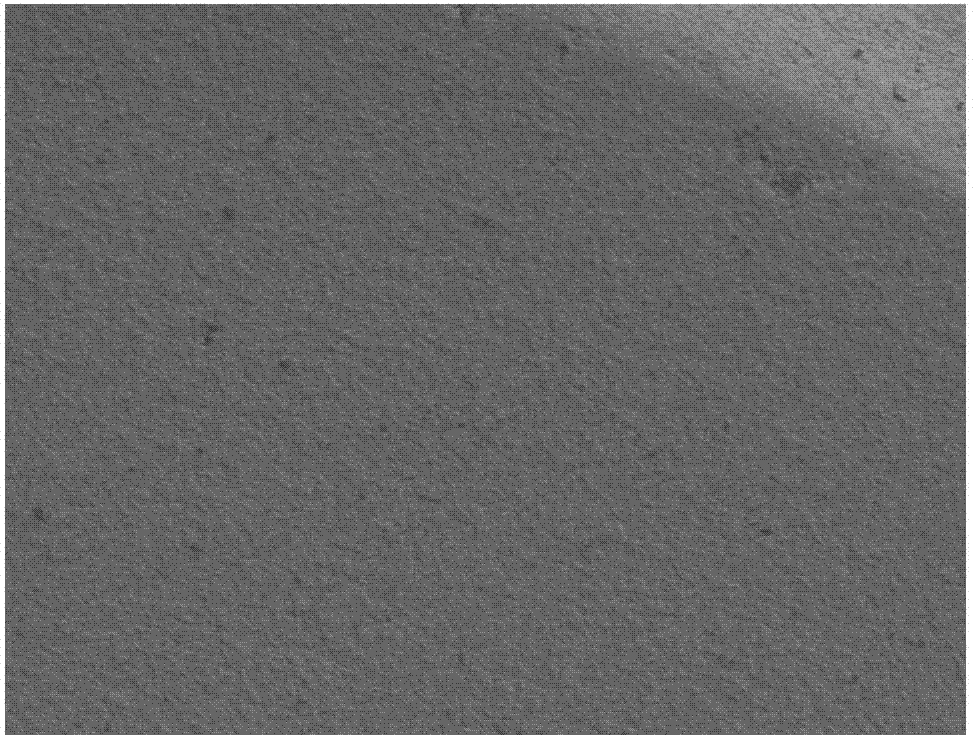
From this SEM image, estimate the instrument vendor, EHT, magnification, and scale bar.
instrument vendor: Hitachi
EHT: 2 kV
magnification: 1 K X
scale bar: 20000 nm